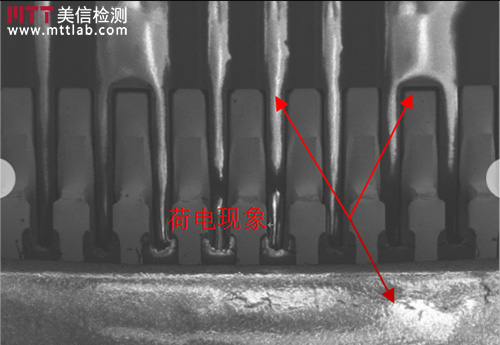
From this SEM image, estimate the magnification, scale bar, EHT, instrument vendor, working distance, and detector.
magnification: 0.03 K X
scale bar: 1000000 nm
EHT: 5 kV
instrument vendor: Hitachi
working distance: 24.6 mm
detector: SE